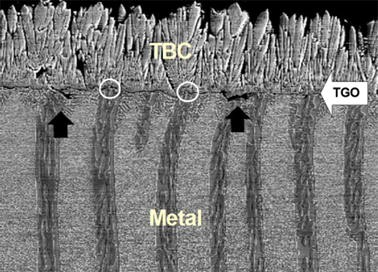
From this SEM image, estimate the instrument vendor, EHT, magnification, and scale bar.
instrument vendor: JEOL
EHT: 10 kV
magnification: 1 K X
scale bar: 20000 nm